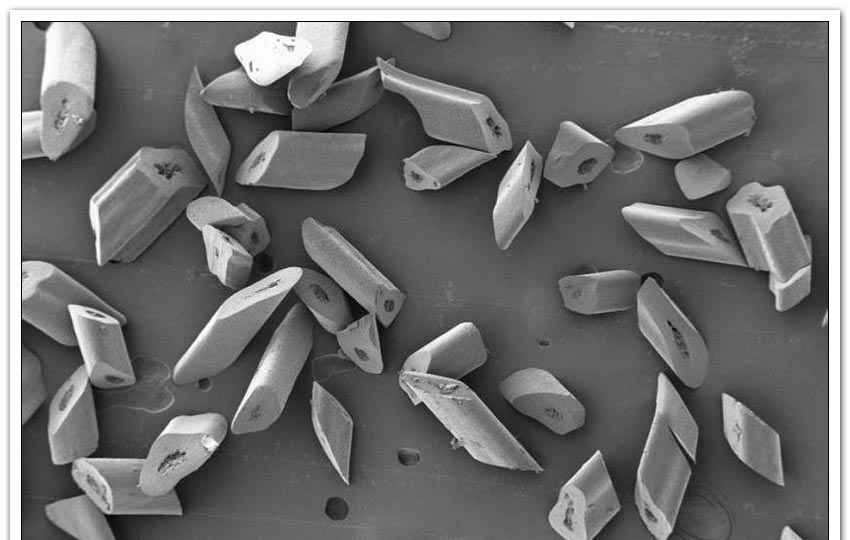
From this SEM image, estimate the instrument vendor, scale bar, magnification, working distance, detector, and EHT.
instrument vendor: Zeiss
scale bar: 100000 nm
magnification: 0.05 K X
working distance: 8.5 mm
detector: SE2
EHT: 3 kV